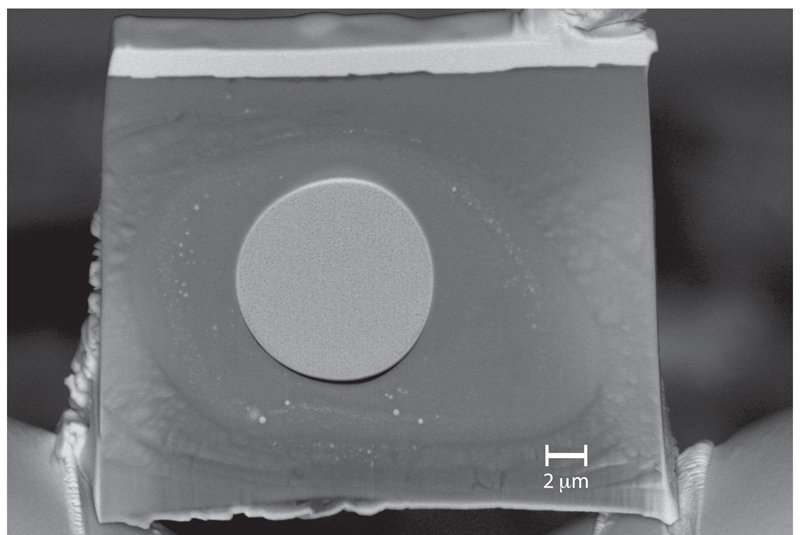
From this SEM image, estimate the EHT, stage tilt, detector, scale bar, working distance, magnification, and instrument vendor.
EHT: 10 kV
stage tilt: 55.5°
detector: ESB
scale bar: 1000 nm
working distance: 5.1 mm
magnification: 2.7 K X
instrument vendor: Zeiss